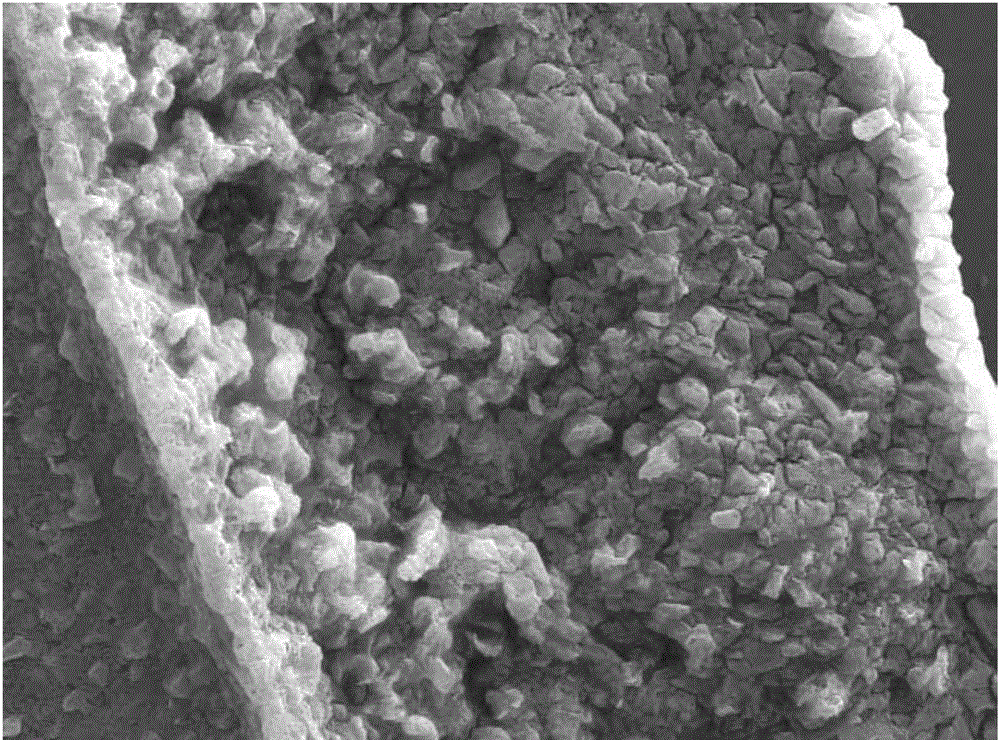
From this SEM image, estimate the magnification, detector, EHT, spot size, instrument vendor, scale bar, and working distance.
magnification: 2 K X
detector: SE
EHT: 20 kV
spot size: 4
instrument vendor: FEI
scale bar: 10000 nm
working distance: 5.7 mm